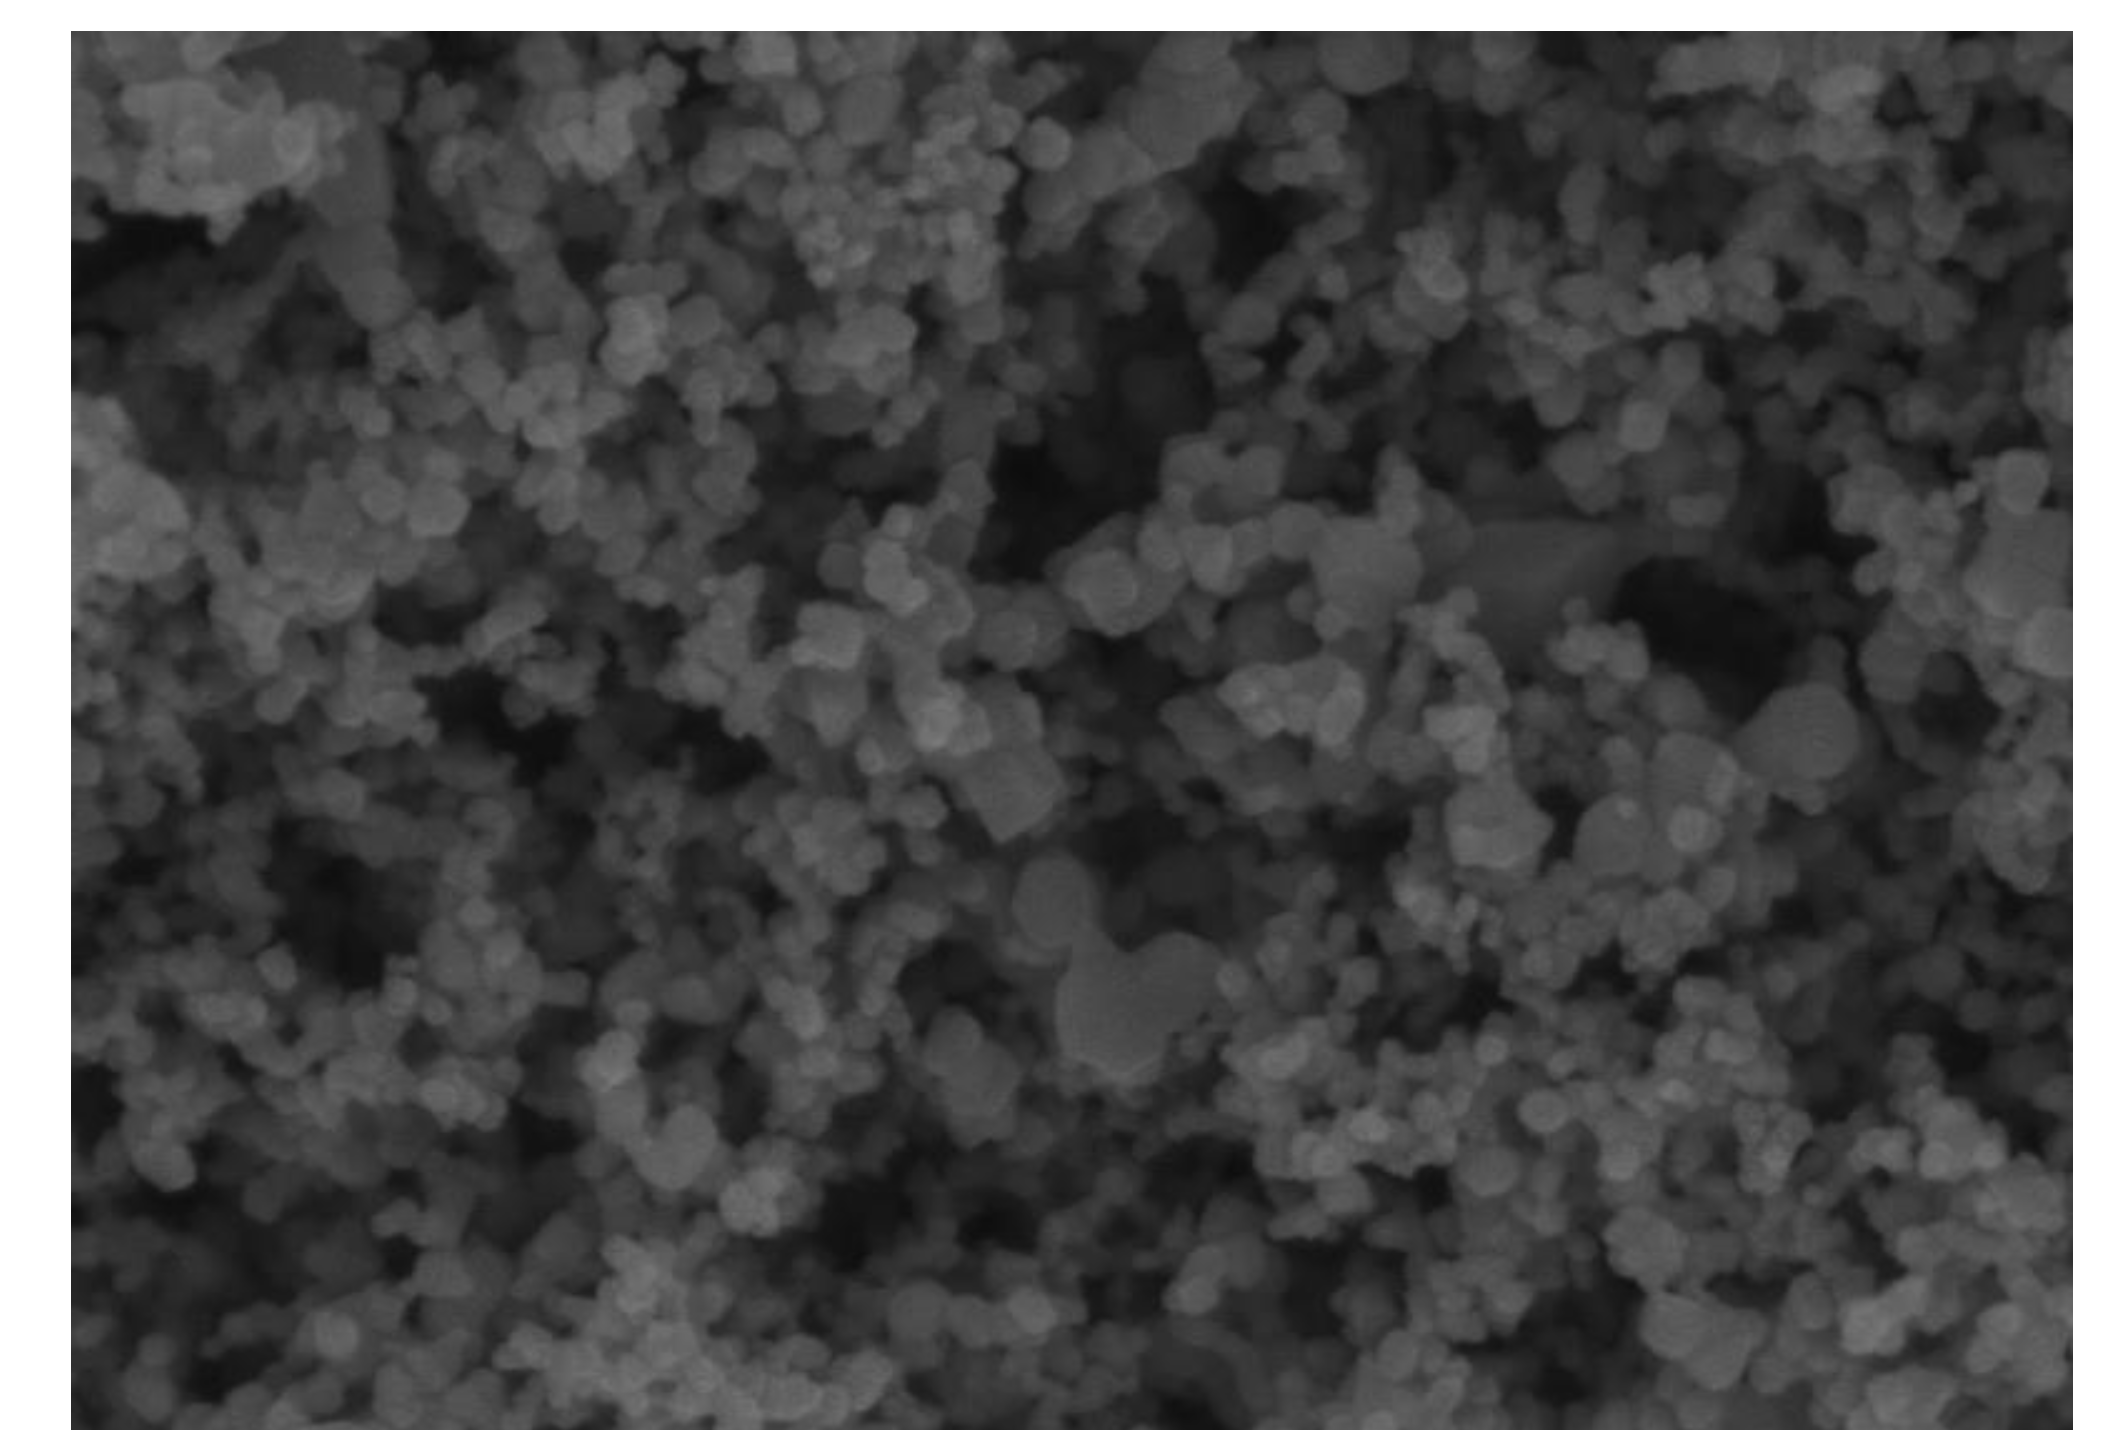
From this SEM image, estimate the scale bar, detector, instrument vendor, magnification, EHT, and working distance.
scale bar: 500 nm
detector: SE(U)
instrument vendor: Hitachi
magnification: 100 K X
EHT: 3 kV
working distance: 5.1 mm